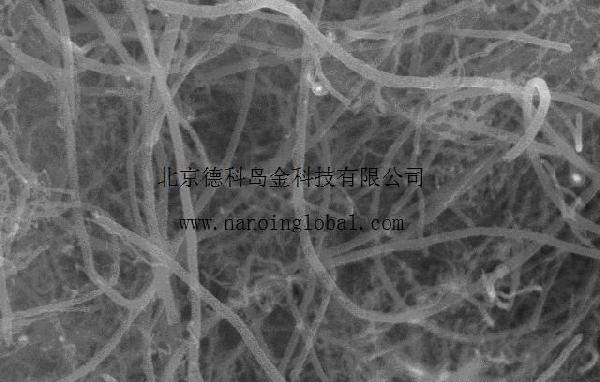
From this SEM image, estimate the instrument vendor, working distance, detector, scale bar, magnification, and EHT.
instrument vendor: Hitachi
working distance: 12.2 mm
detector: SE(M.-150)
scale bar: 1000 nm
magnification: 30 K X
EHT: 15 kV